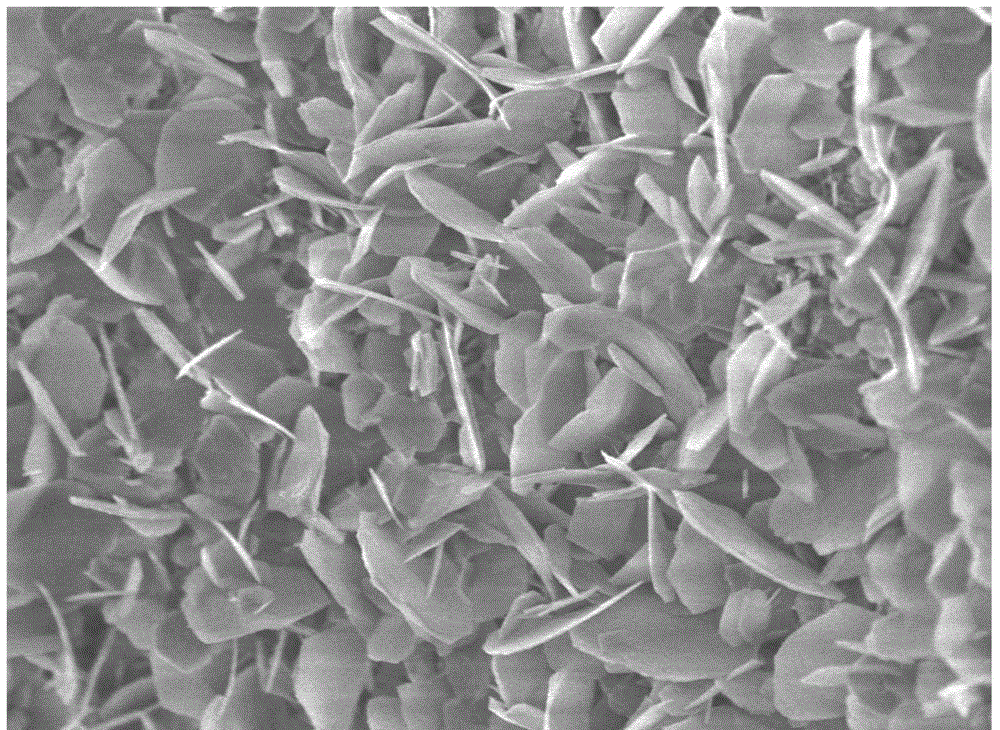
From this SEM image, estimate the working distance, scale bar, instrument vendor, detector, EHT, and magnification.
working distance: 3 mm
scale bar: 1000 nm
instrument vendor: JEOL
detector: UED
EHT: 3 kV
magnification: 10 K X